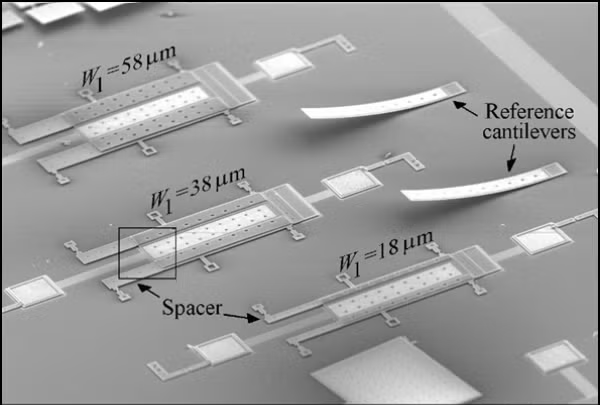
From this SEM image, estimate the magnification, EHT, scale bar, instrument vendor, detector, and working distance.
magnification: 0.1 K X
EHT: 15 kV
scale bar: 500000 nm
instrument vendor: JEOL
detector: SE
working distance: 30.5 mm